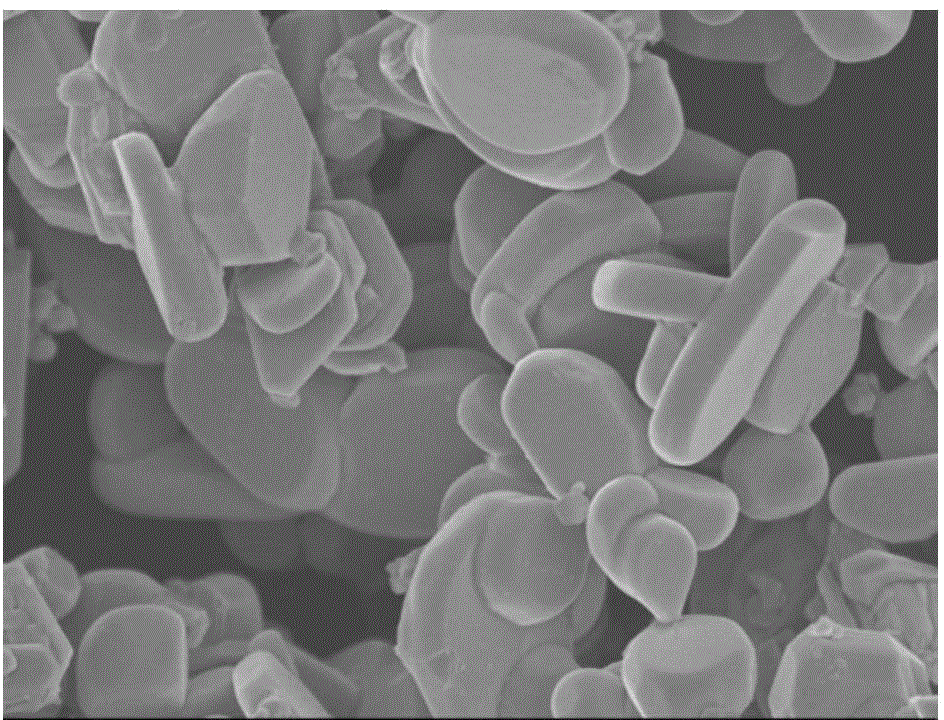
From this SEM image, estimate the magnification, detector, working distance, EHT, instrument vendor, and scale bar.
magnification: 9.5 K X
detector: SEI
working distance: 7.8 mm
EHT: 5 kV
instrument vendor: JEOL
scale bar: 1000 nm